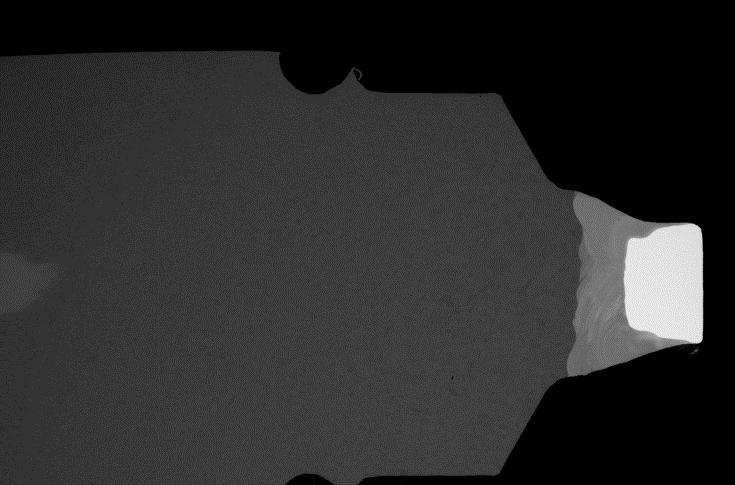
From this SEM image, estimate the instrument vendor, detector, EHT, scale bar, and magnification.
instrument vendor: JEOL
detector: BEC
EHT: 20 kV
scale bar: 500000 nm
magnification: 0.03 K X